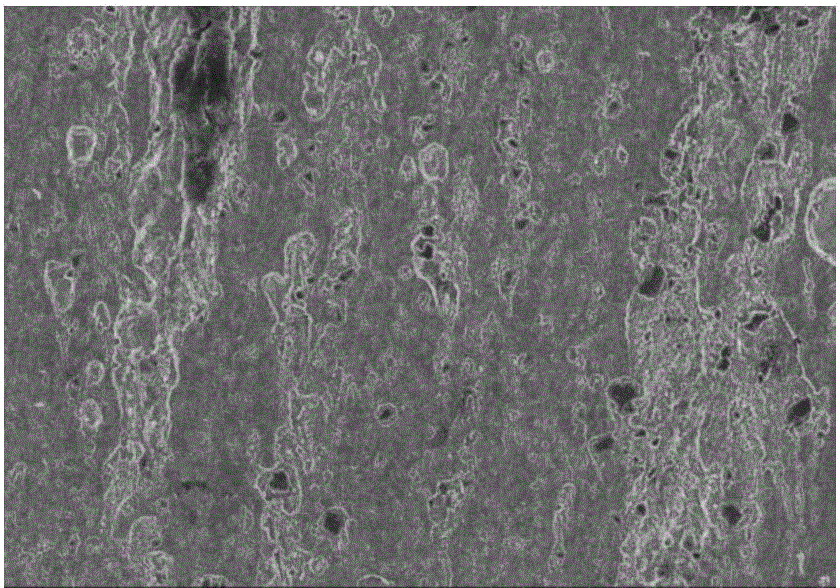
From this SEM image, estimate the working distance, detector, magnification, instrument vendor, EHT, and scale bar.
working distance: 11 mm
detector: SE(U)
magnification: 5 K X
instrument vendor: Hitachi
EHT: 3 kV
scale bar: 10000 nm